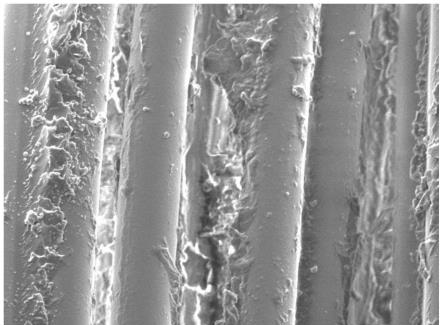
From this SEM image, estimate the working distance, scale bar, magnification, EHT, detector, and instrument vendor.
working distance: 16.7 mm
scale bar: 10000 nm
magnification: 2 K X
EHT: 5 kV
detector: LED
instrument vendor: JEOL